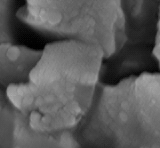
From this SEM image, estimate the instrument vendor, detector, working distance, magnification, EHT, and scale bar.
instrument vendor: JEOL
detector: SEI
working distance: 5.1 mm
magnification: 50 K X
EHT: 10 kV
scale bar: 100 nm